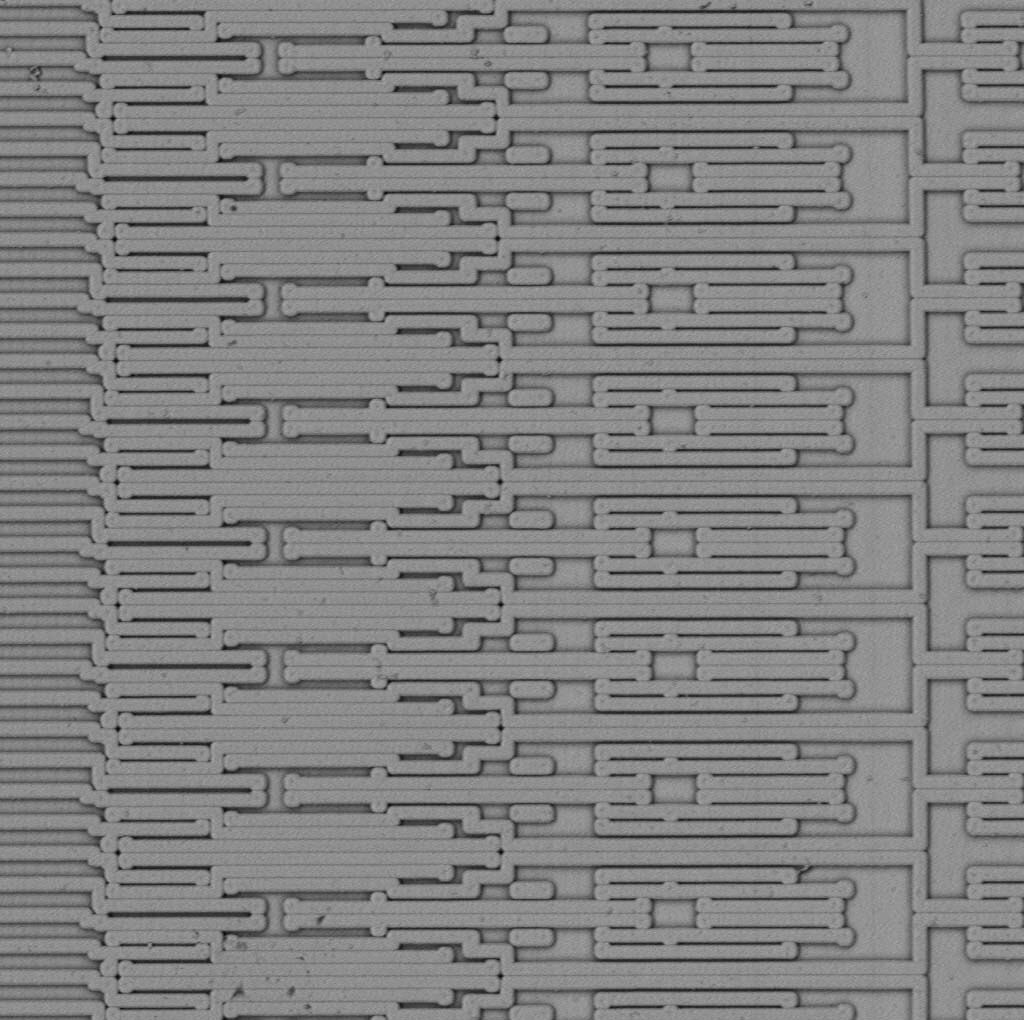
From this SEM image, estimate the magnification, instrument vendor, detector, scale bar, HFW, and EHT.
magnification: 2.05 K X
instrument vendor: Thermo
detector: BSD Full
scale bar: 30000 nm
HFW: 131 µm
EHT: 5 kV